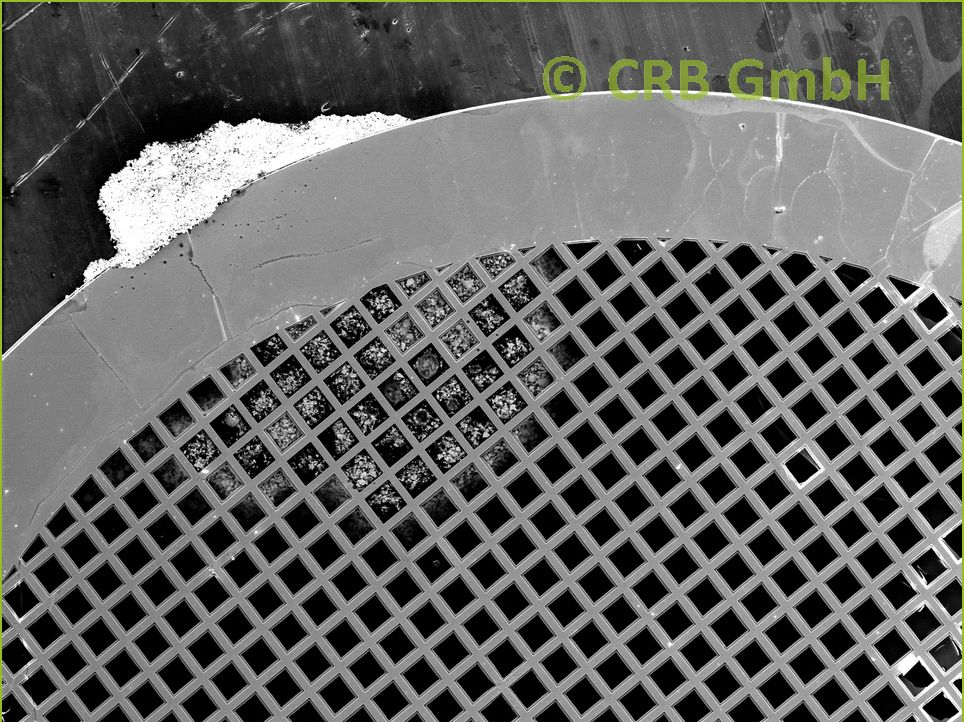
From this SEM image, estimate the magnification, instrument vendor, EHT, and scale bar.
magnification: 0.062 K X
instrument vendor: Hitachi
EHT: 20 kV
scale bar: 200000 nm